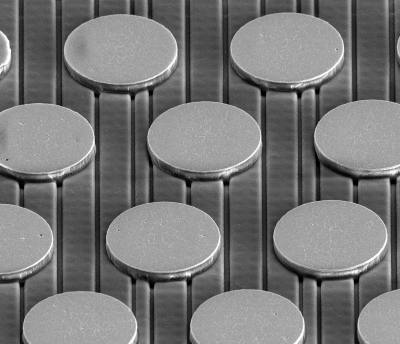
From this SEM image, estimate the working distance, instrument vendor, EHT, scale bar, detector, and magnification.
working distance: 12.1 mm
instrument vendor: FEI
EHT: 20 kV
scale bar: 100000 nm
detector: ETD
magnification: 0.8 K X